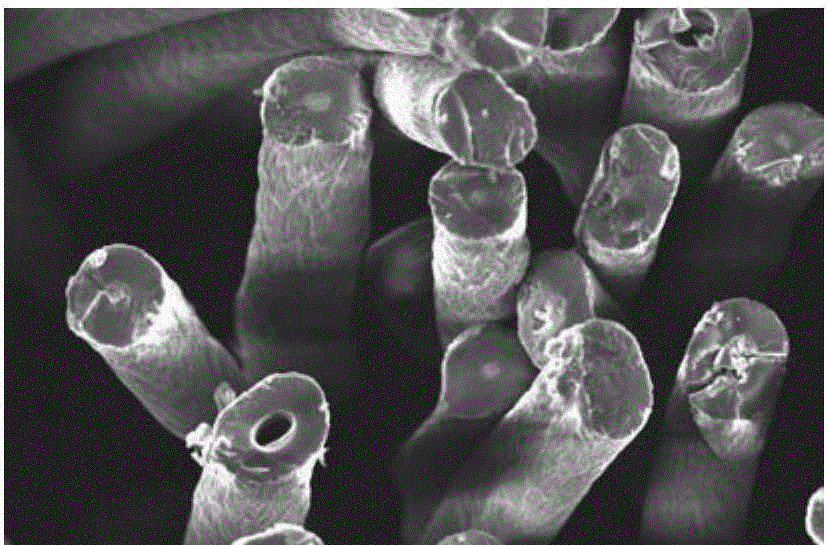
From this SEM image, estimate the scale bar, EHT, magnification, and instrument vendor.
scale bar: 10000 nm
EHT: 8 kV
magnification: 1 K X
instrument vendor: JEOL